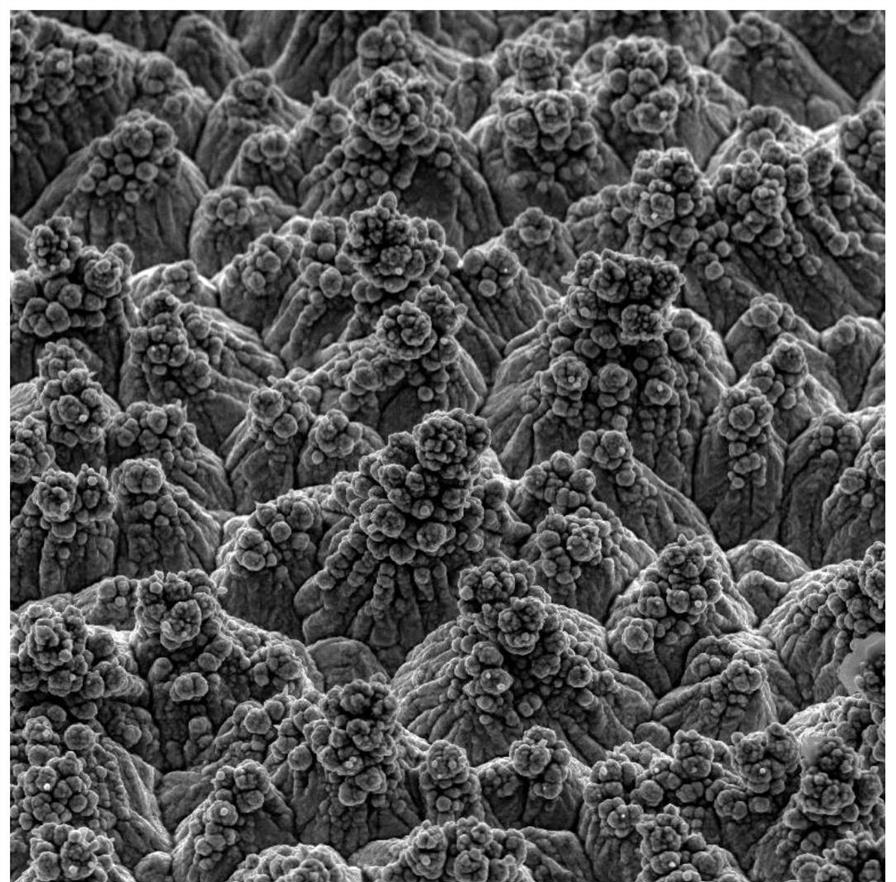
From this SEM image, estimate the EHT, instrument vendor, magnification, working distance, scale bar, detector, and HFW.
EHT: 10 kV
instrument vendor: Tescan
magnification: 5 K X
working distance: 16.58 mm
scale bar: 10000 nm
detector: SE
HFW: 41.5 µm